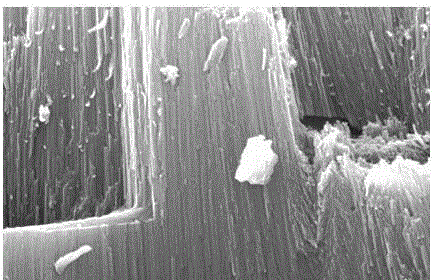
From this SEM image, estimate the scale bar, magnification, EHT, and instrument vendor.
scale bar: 1000 nm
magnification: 10 K X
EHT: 25 kV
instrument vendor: JEOL